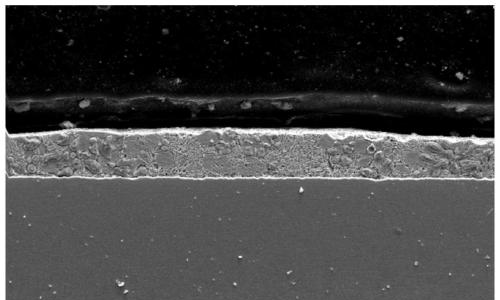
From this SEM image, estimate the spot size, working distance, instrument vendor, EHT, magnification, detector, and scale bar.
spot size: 4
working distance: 6.6 mm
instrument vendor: FEI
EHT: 20 kV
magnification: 1 K X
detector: SE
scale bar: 50000 nm